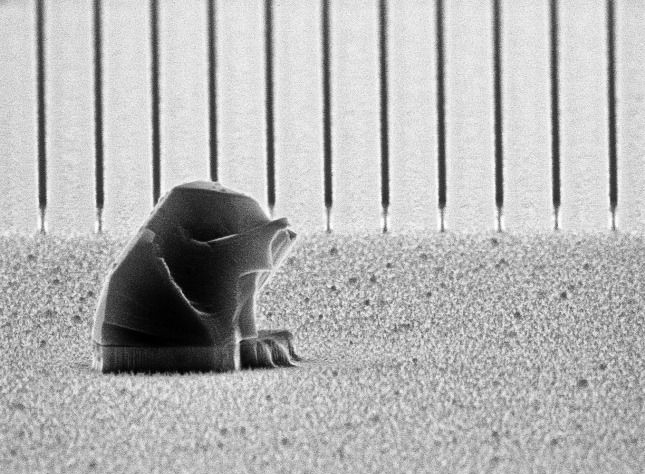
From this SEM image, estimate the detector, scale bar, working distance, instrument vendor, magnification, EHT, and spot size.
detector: SE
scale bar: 2000 nm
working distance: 21.8 mm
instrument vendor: FEI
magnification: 14.014 K X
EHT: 10 kV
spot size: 3